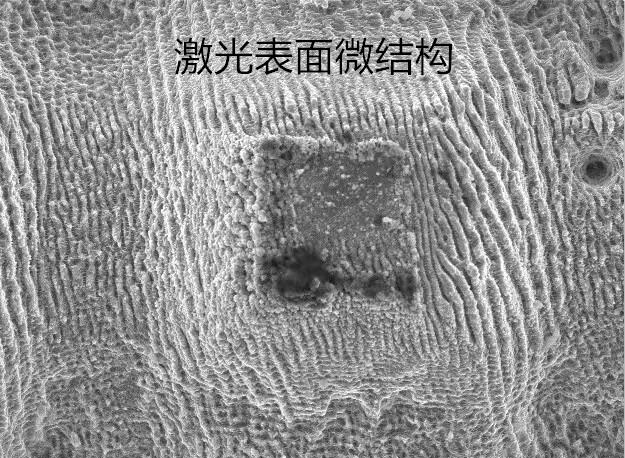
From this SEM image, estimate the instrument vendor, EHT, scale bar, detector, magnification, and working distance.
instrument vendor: JEOL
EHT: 15 kV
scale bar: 10000 nm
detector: SEI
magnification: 2 K X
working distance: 12 mm